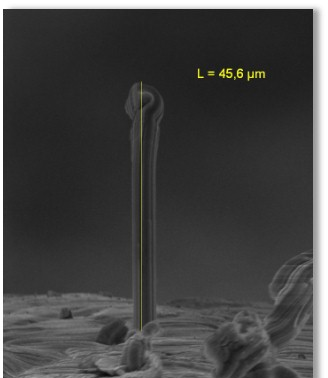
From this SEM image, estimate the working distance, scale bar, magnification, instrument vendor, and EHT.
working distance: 40 mm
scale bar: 10000 nm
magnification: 1.7 K X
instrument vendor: Tescan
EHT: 20 kV